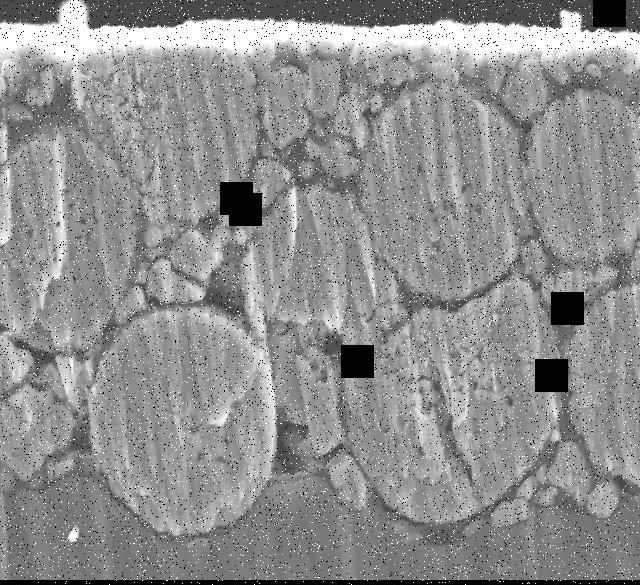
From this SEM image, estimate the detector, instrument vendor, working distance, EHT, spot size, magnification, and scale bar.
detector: SEI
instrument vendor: JEOL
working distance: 8 mm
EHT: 15 kV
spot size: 45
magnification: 3 K X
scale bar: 5000 nm